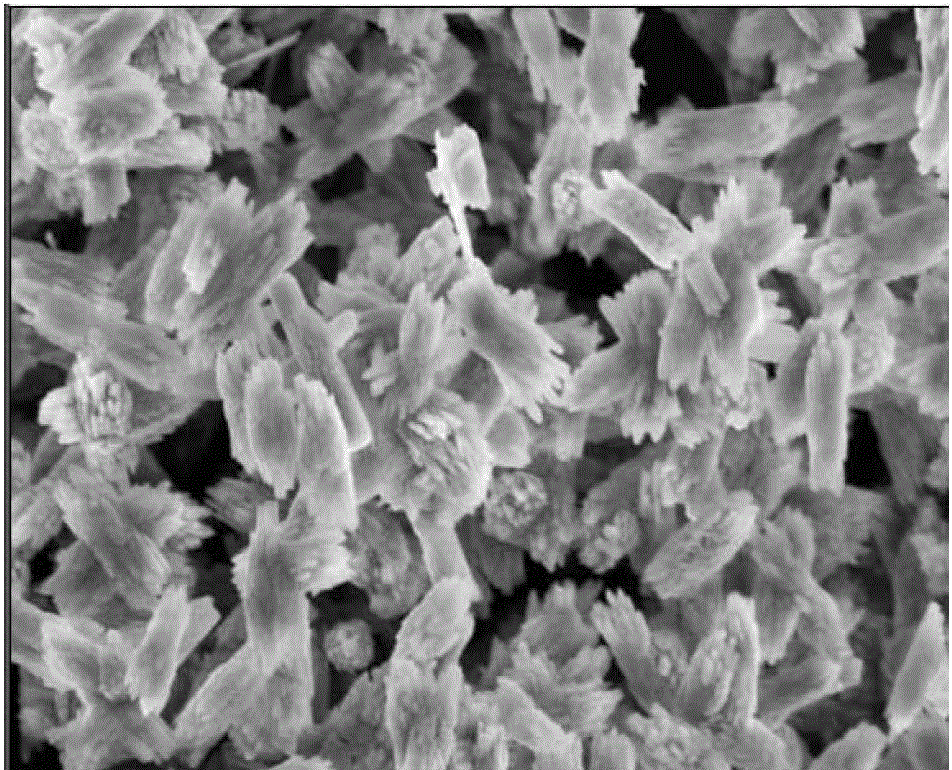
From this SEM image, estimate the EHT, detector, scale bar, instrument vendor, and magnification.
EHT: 5 kV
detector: SE(M)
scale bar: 5000 nm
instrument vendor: Hitachi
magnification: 10 K X